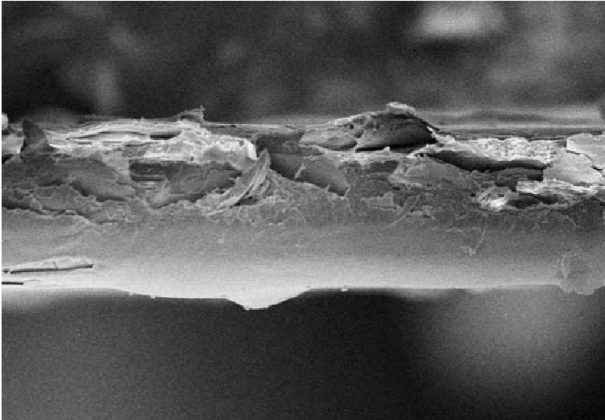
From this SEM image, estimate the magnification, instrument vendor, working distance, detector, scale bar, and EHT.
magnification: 0.6 K X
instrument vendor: Hitachi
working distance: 7.4 mm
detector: SE(U)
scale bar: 50000 nm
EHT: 5 kV